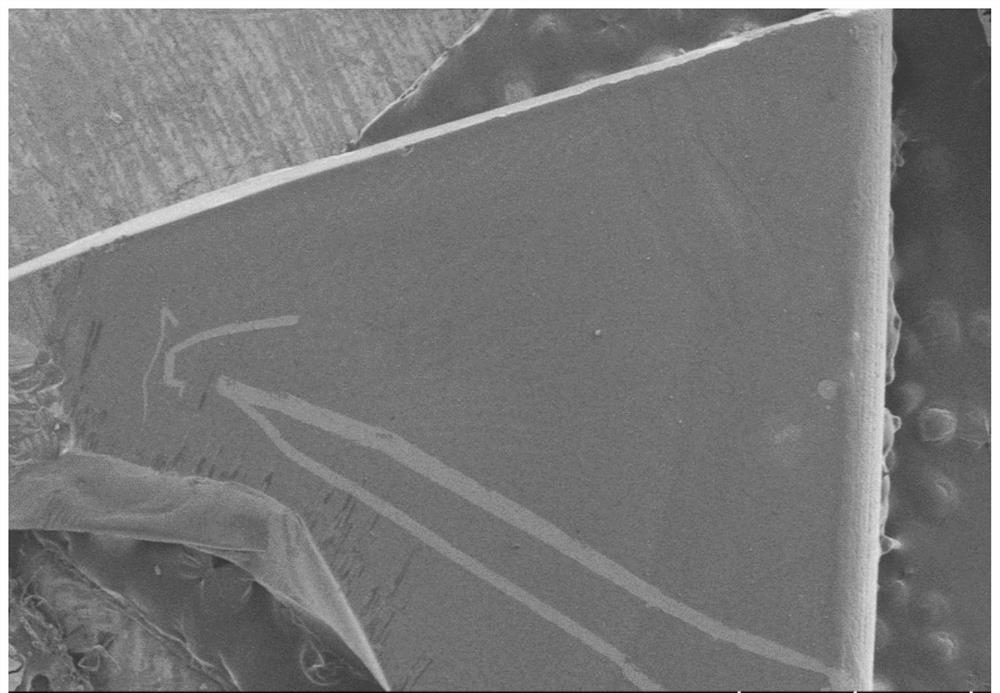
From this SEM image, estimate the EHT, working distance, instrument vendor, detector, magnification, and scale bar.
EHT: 3 kV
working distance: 8.9 mm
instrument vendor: Hitachi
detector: LM(L)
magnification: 0.03 K X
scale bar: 1000000 nm